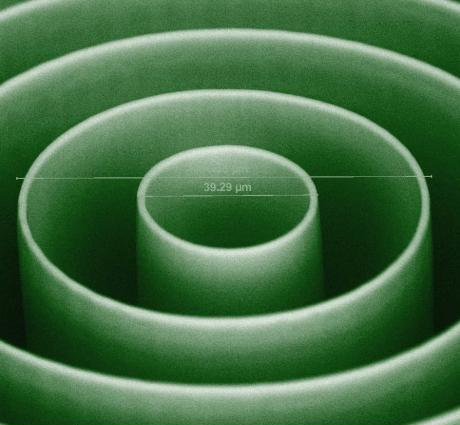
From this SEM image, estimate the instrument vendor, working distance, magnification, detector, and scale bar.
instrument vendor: FEI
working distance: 12.3 mm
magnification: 2.355 K X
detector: BSED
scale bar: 50000 nm